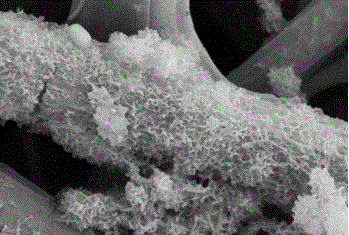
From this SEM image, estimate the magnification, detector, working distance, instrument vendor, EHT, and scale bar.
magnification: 11 K X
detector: SE2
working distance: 4.4 mm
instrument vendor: Zeiss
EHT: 10 kV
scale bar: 1000 nm